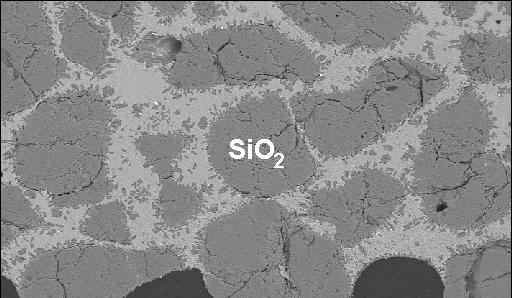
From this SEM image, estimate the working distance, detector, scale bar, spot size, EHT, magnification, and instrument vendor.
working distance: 10.2 mm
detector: BSE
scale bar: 200000 nm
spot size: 5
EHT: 20 kV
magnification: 0.1 K X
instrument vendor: FEI